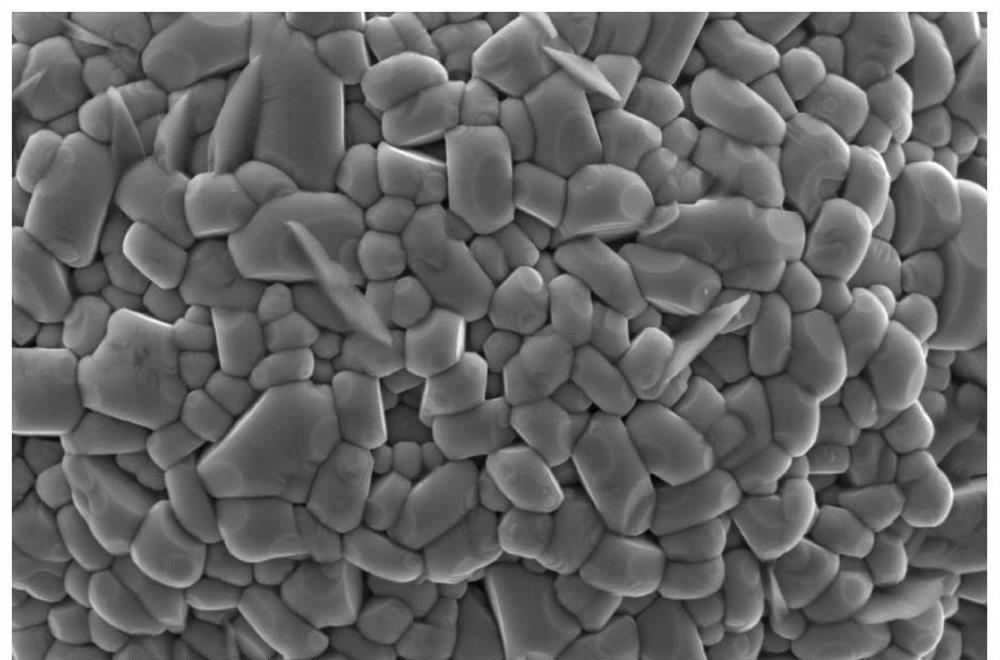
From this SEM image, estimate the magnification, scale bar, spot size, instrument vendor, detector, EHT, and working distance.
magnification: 50 K X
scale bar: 3000 nm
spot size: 3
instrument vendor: FEI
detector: ETD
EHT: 5 kV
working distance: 10.2 mm